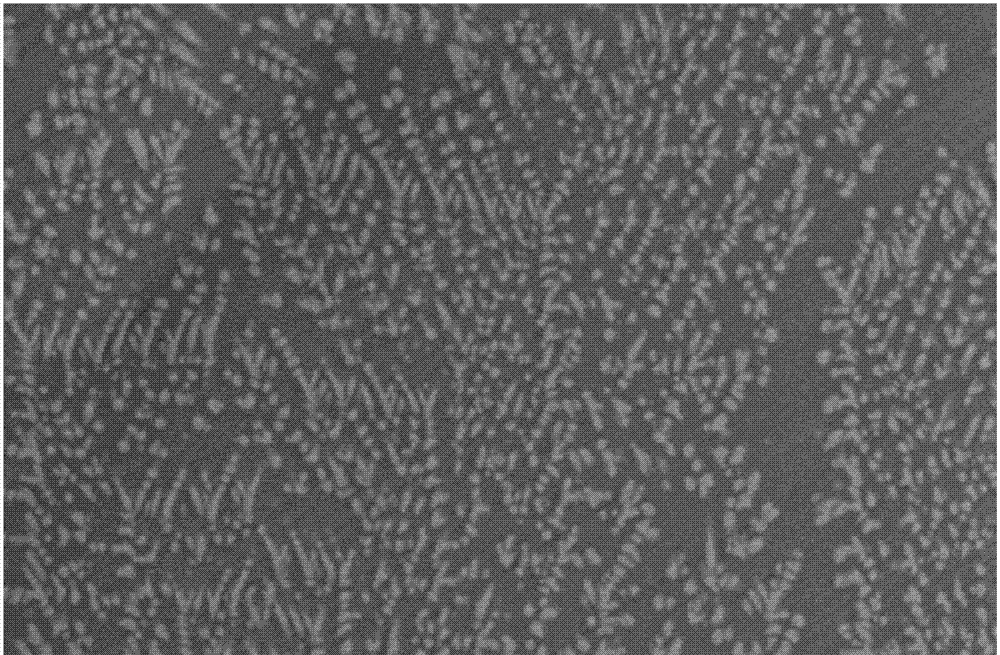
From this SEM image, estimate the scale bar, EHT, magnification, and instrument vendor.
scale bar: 10000 nm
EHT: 20 kV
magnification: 1 K X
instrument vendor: JEOL